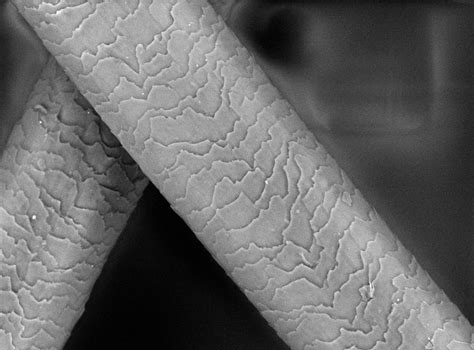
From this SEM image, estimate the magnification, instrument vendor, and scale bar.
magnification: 1 K X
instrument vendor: Hitachi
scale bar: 100000 nm